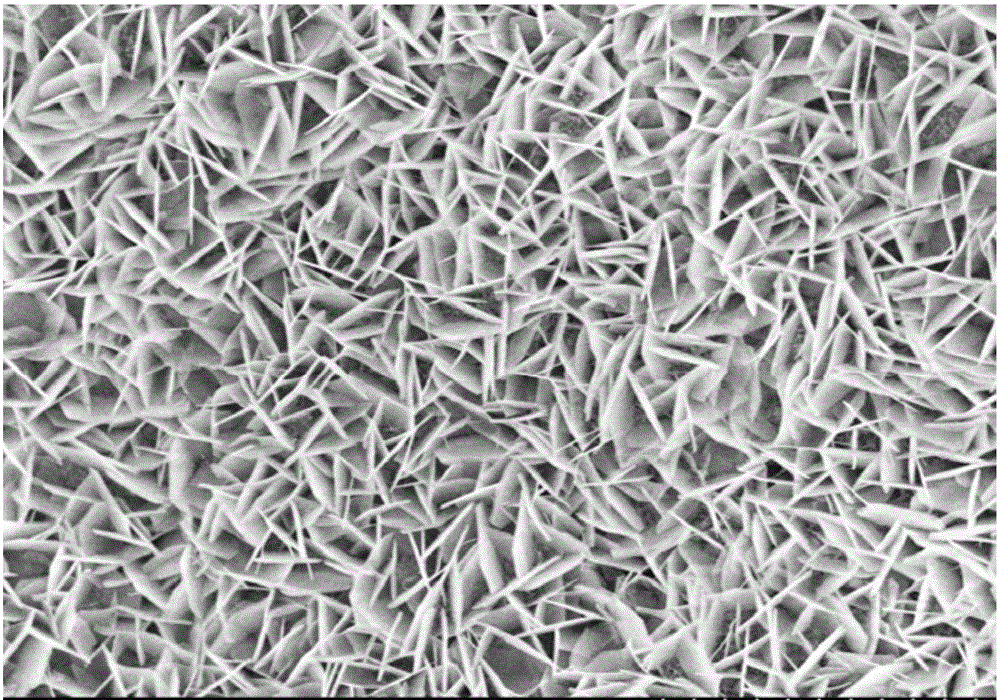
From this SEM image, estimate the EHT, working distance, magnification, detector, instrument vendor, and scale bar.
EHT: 5 kV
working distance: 8 mm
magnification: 8 K X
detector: SE(M,LA0)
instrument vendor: Hitachi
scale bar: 5000 nm